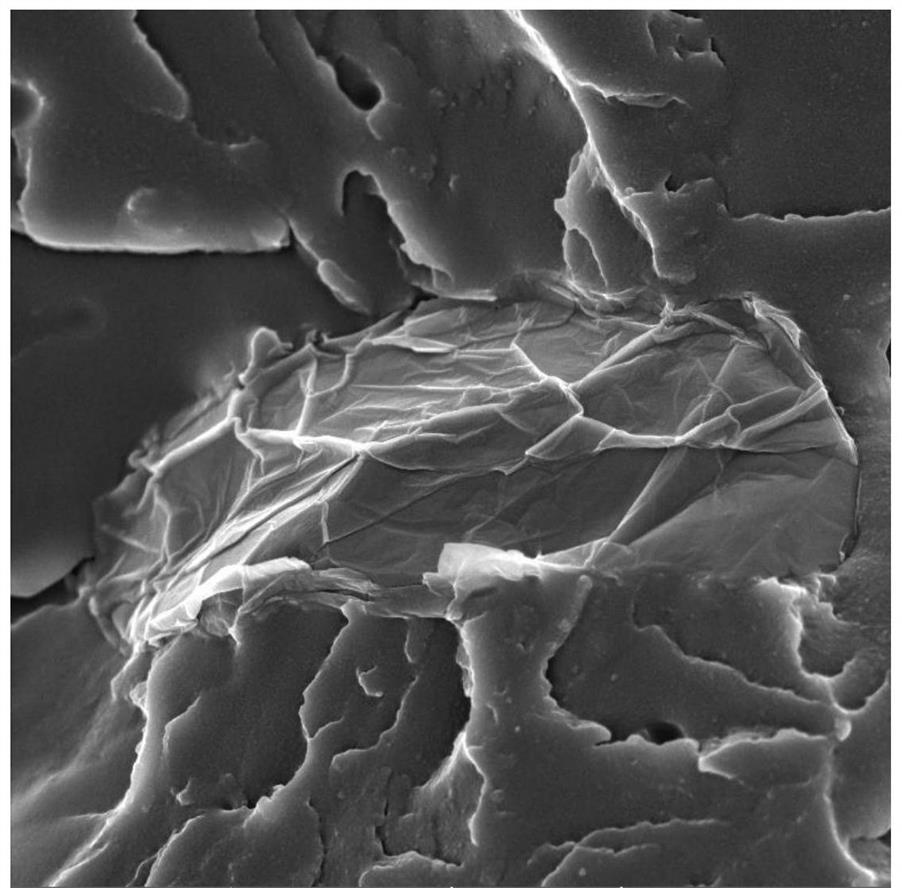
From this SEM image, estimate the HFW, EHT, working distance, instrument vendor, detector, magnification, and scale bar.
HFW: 10.4 µm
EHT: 5 kV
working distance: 4.98 mm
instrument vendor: Tescan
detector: In-Beam SE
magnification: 20 K X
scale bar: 2000 nm